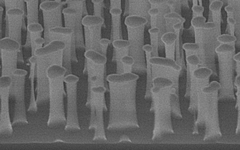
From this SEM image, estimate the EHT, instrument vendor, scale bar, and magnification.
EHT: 10 kV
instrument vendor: JEOL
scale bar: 500 nm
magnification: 60 K X